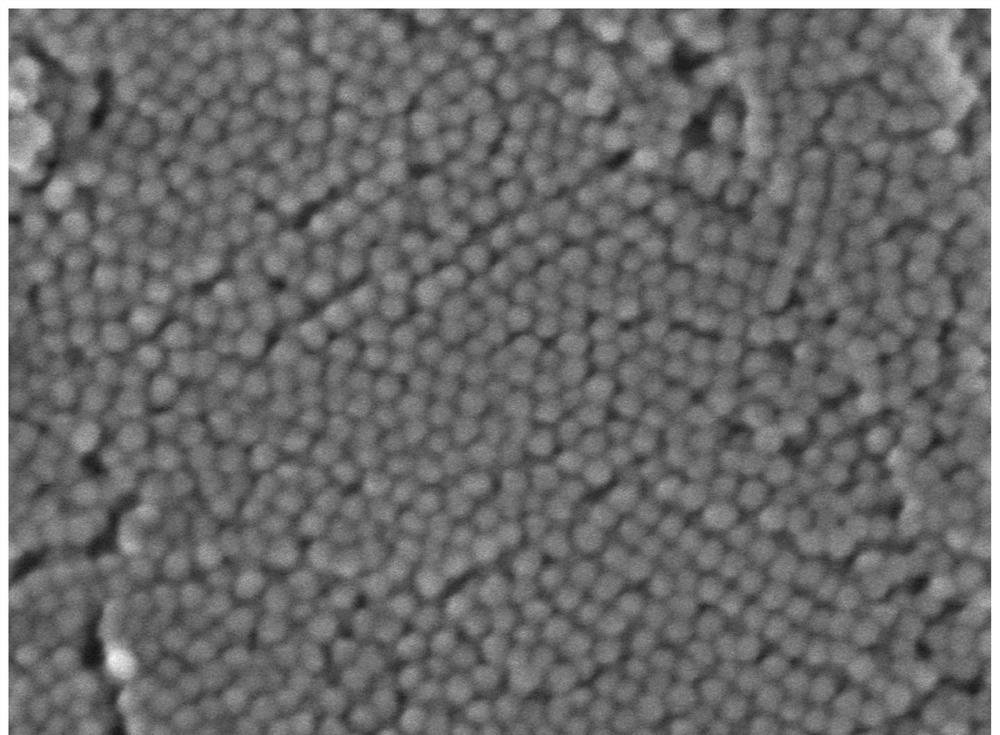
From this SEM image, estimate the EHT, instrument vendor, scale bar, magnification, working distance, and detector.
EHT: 1 kV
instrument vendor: JEOL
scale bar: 100 nm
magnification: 150 K X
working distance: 3.1 mm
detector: UED